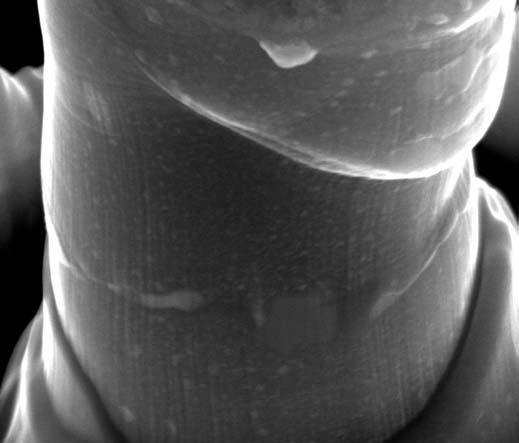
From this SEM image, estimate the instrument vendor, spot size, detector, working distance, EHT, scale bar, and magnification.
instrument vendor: FEI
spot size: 5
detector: ETD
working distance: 9.9 mm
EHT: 20 kV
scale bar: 2000 nm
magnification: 35 K X